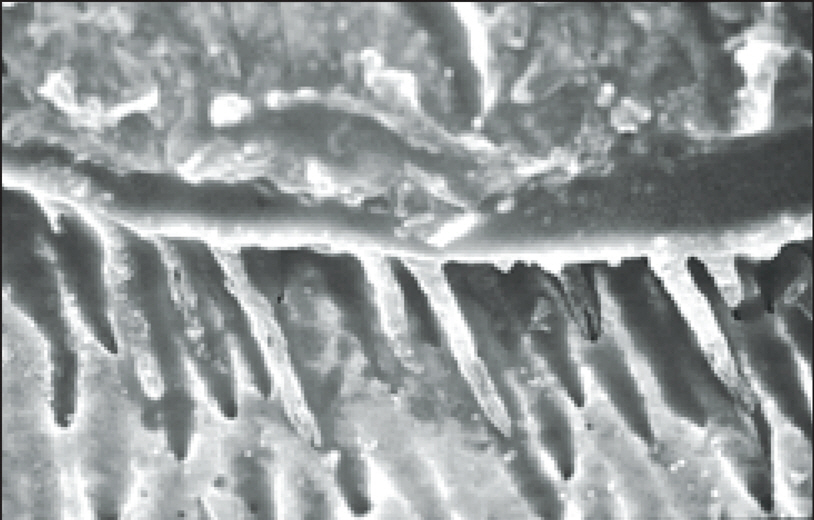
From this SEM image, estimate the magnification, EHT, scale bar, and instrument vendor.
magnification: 1 K X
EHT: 20 kV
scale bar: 50000 nm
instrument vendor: Hitachi